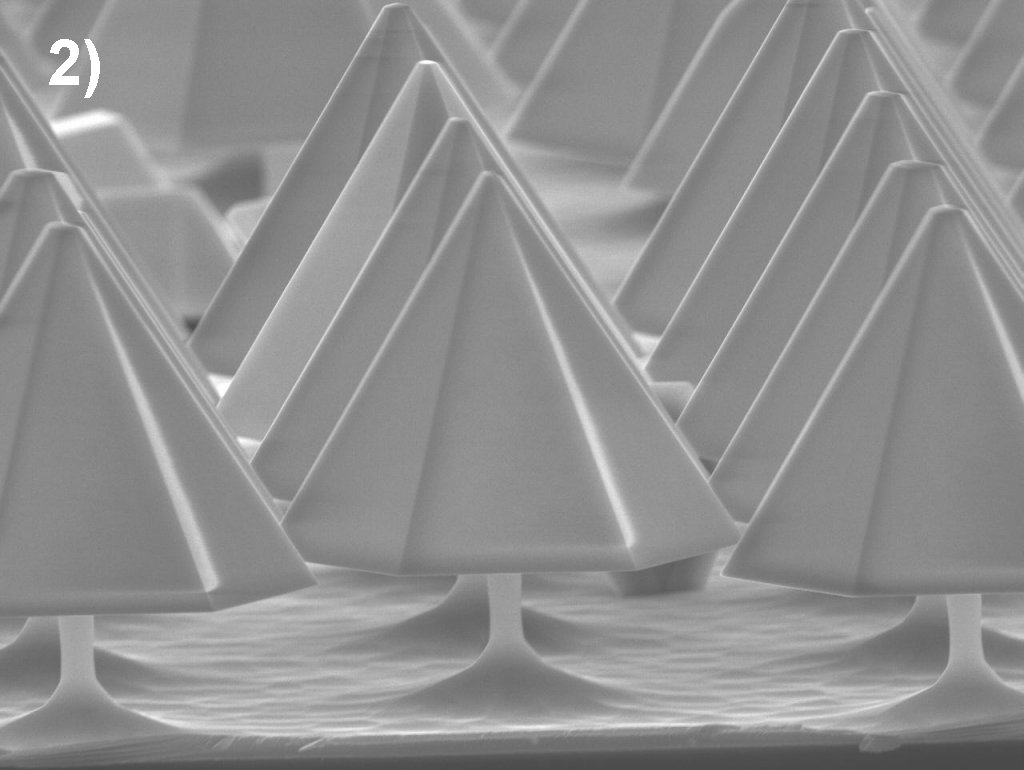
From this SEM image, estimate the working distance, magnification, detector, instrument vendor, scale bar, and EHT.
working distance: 10 mm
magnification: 4 K X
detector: SEI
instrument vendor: JEOL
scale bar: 1000 nm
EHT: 15 kV